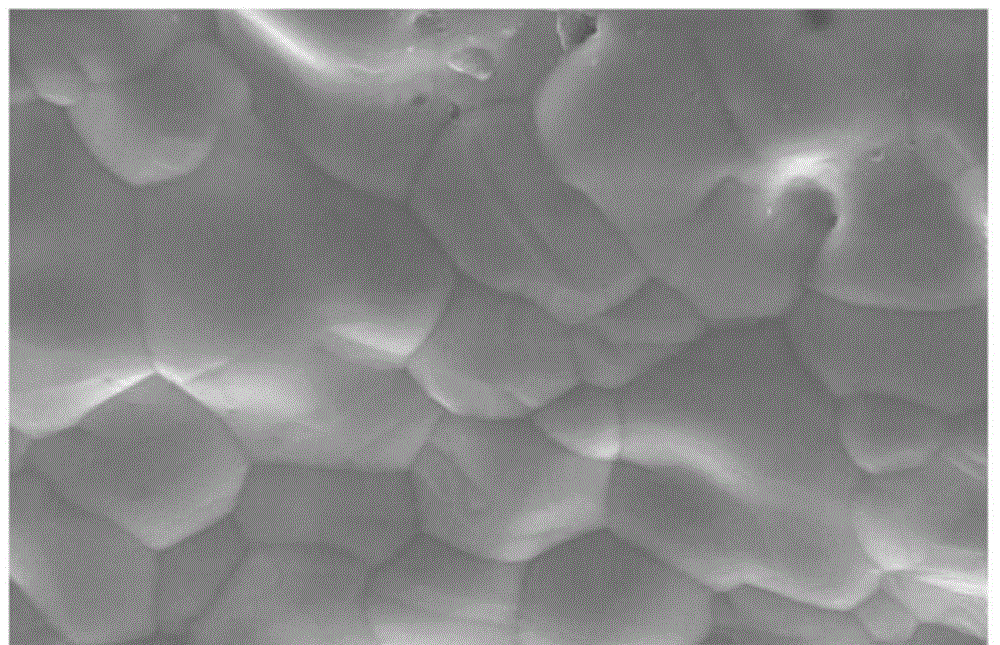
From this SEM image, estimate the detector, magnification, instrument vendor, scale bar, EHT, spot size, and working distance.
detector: TLD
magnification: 10 K X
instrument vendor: FEI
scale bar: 5000 nm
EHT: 20 kV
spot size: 4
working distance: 7.4 mm